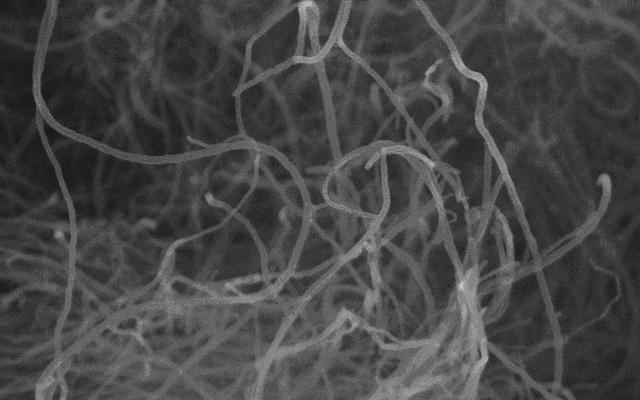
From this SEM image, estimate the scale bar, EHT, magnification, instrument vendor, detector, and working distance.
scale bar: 1000 nm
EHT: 15 kV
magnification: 30 K X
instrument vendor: Hitachi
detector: SE(M,-150)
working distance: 12.2 mm